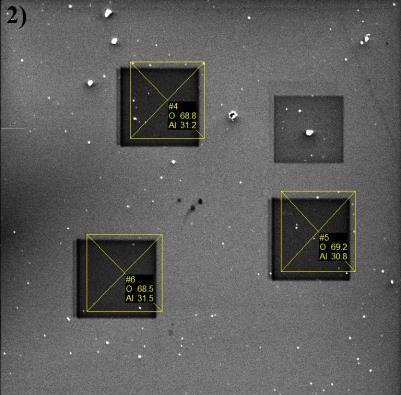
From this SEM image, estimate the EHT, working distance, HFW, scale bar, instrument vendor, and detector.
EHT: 5 kV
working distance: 15.37 mm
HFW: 178 µm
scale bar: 50000 nm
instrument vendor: Tescan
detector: SE